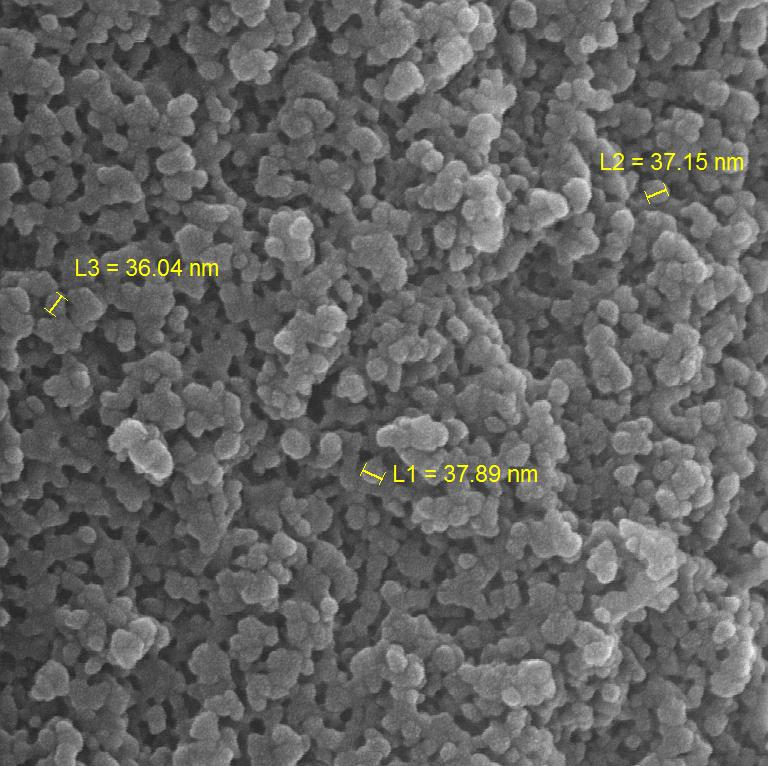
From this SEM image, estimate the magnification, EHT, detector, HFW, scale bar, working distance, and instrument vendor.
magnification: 150 K X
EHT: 15 kV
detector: InBeam SE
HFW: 1.38 µm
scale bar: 200 nm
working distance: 5.07 mm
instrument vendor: Tescan